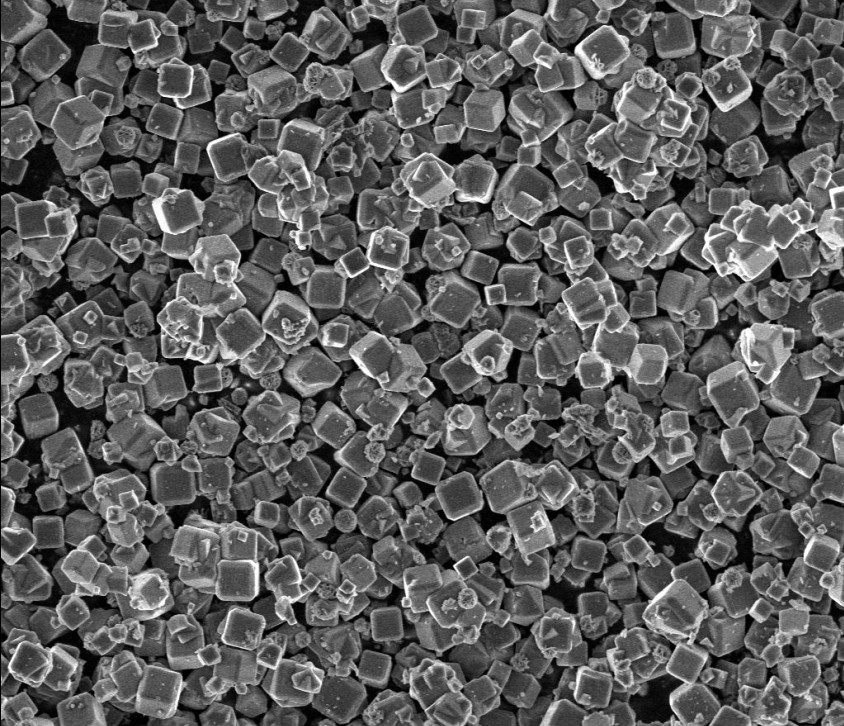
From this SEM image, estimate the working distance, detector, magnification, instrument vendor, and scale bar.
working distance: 4.9 mm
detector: TLD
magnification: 2 K X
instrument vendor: FEI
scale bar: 30000 nm